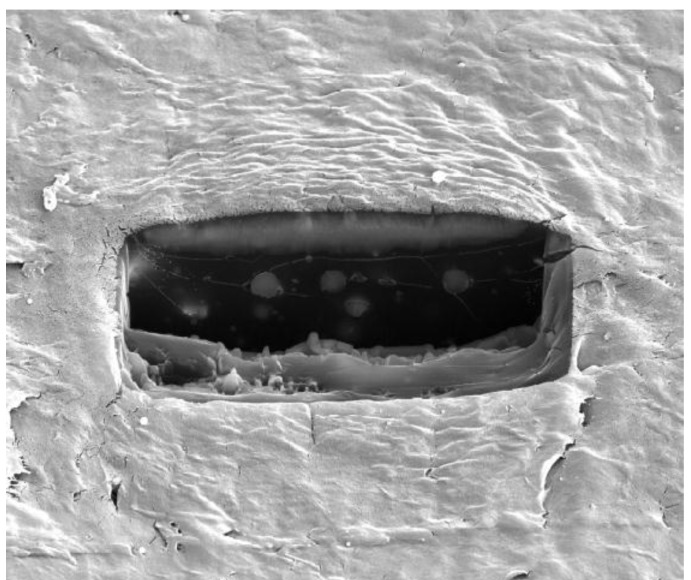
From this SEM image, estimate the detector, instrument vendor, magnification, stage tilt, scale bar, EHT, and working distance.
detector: ETD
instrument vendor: FEI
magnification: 1.5 K X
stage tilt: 52°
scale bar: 40000 nm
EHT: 20 kV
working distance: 5 mm